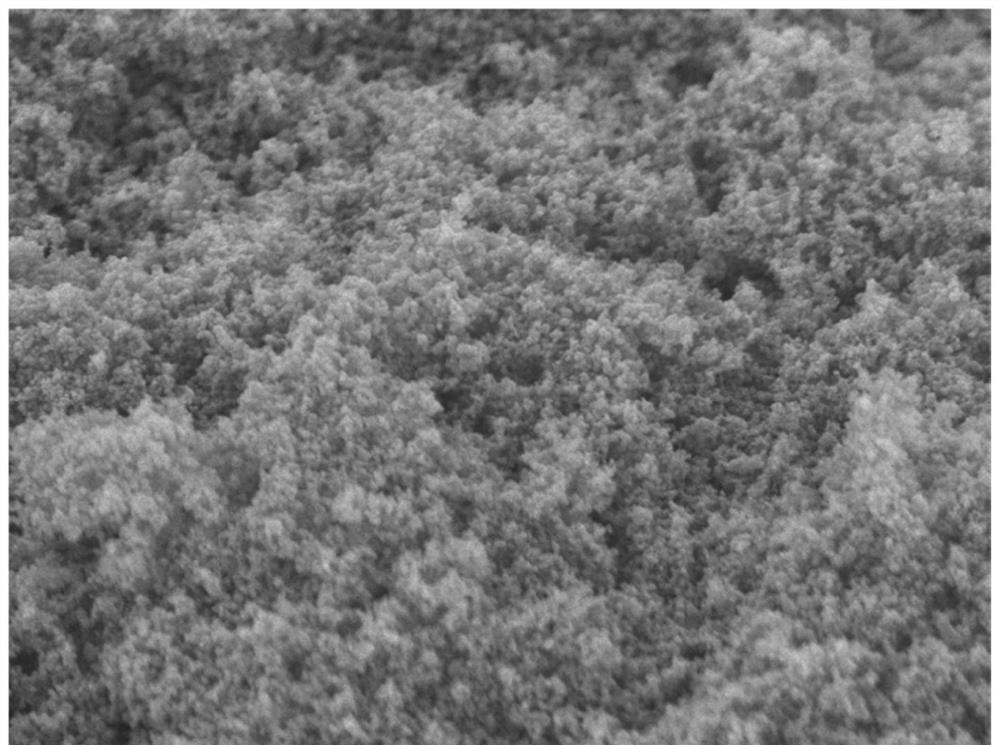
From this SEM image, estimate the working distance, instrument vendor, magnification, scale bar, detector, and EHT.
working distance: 5.9 mm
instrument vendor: JEOL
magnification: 20 K X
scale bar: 1000 nm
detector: SEI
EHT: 10 kV